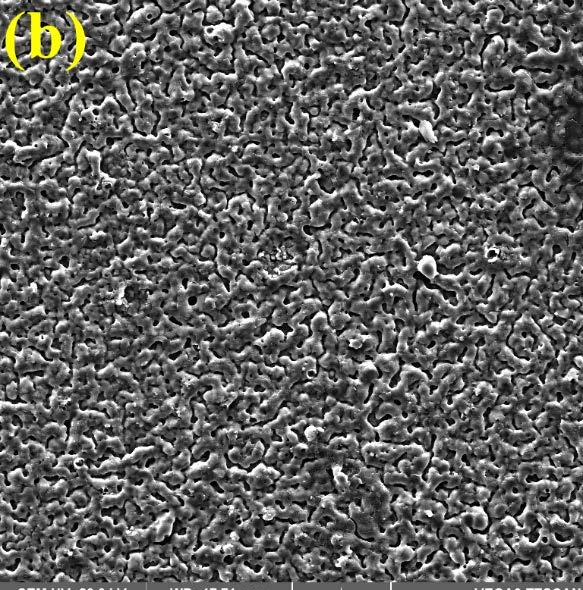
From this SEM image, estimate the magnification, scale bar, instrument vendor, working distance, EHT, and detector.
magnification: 0.703 K X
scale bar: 50000 nm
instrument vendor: Tescan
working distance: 17.71 mm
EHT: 20 kV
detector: SE